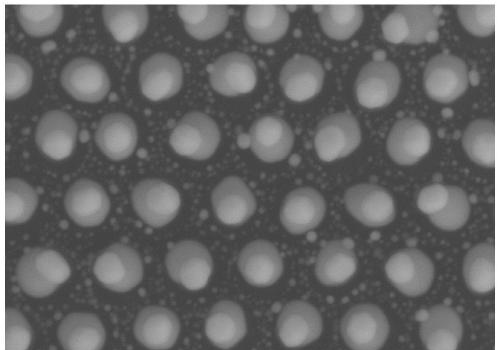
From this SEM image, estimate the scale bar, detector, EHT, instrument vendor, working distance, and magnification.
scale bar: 500 nm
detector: SE(TUL)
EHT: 10 kV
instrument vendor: Hitachi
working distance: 13.5 mm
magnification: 60 K X